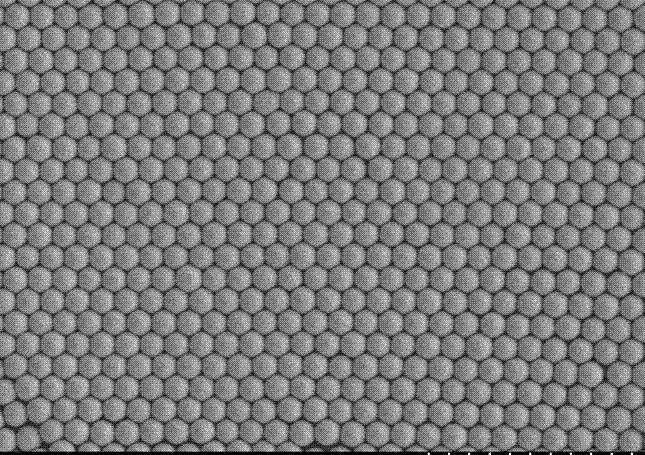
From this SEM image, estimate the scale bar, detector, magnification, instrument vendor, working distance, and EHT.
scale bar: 2000 nm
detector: SE(M)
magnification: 20 K X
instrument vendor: Hitachi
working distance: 9.3 mm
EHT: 5 kV